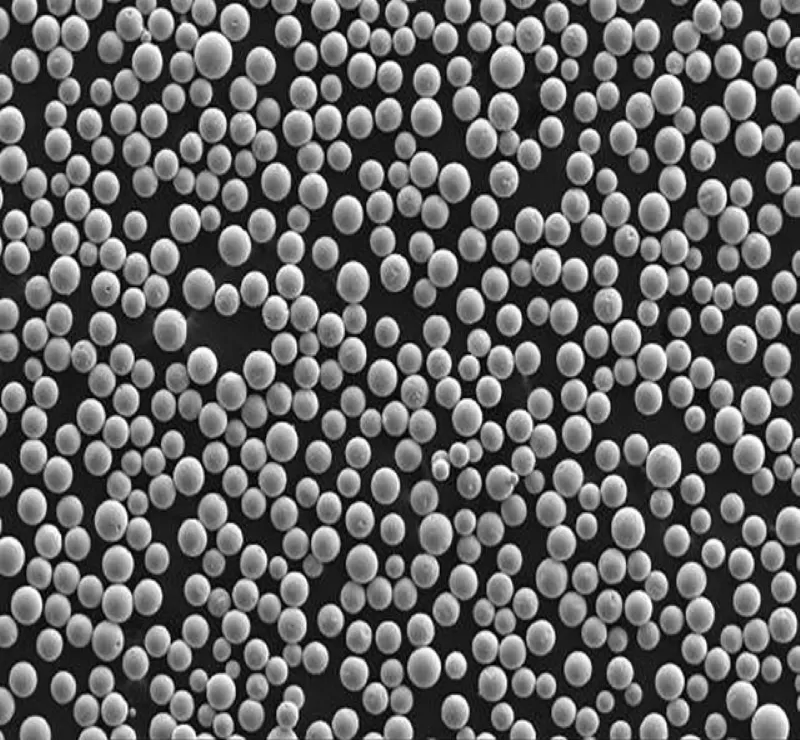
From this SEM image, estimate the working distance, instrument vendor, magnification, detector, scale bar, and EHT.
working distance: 12 mm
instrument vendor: JEOL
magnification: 0.1 K X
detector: SEI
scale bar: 100000 nm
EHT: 20 kV